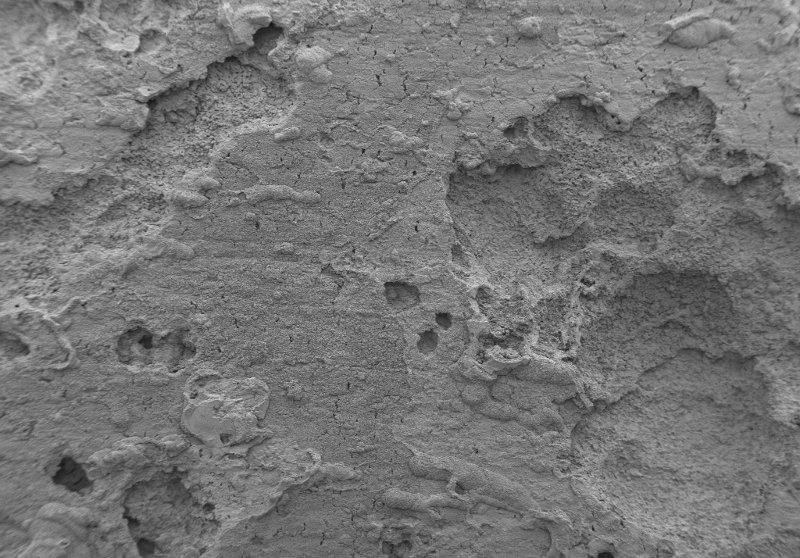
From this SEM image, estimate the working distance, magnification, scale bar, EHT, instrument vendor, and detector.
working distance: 11.1 mm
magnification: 0.025 K X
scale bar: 200000 nm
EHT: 5 kV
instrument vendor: Zeiss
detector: SE2